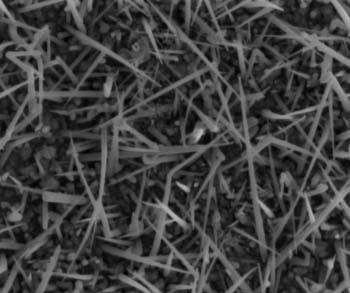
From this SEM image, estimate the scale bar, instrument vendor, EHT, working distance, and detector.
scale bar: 1000 nm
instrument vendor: FEI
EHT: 2 kV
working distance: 7 mm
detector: ETD